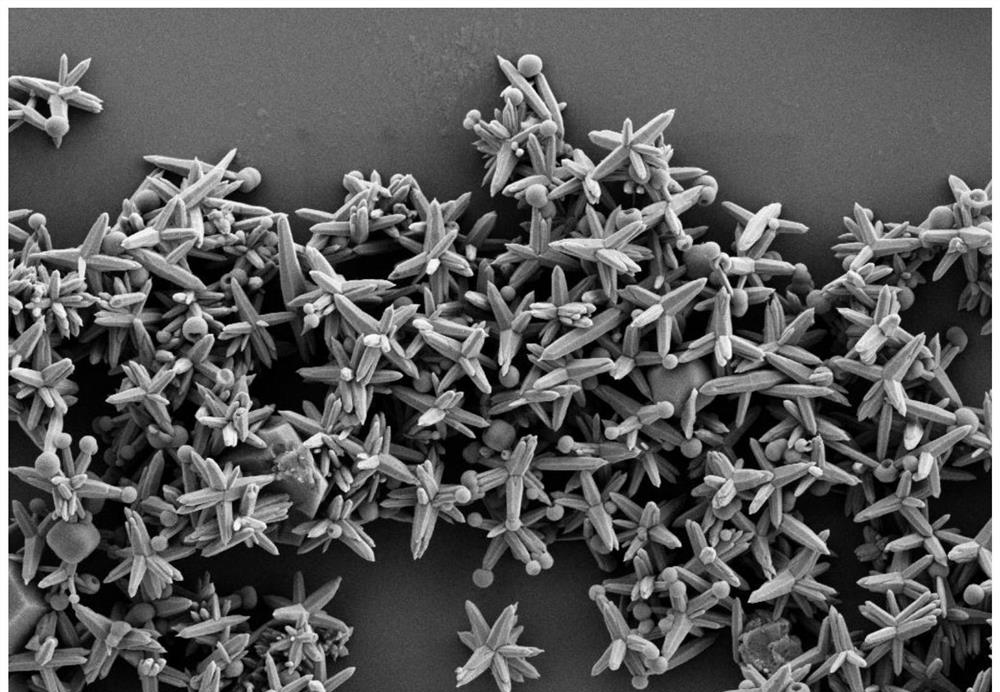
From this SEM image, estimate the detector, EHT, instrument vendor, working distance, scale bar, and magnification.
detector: SE2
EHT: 3 kV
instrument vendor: Zeiss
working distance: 8.4 mm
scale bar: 1000 nm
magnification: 5 K X